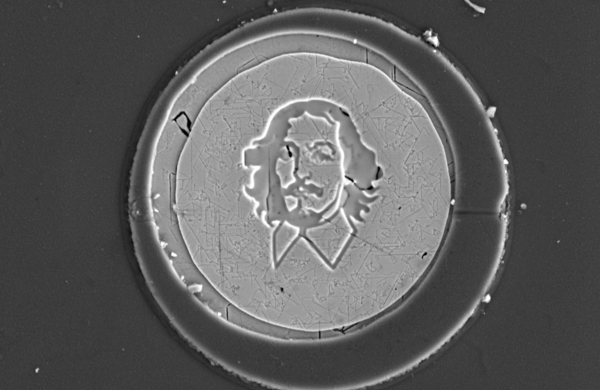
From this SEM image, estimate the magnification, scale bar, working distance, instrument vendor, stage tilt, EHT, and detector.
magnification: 10 K X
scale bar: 5000 nm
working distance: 7.1 mm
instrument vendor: FEI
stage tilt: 0°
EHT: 5 kV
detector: ETD